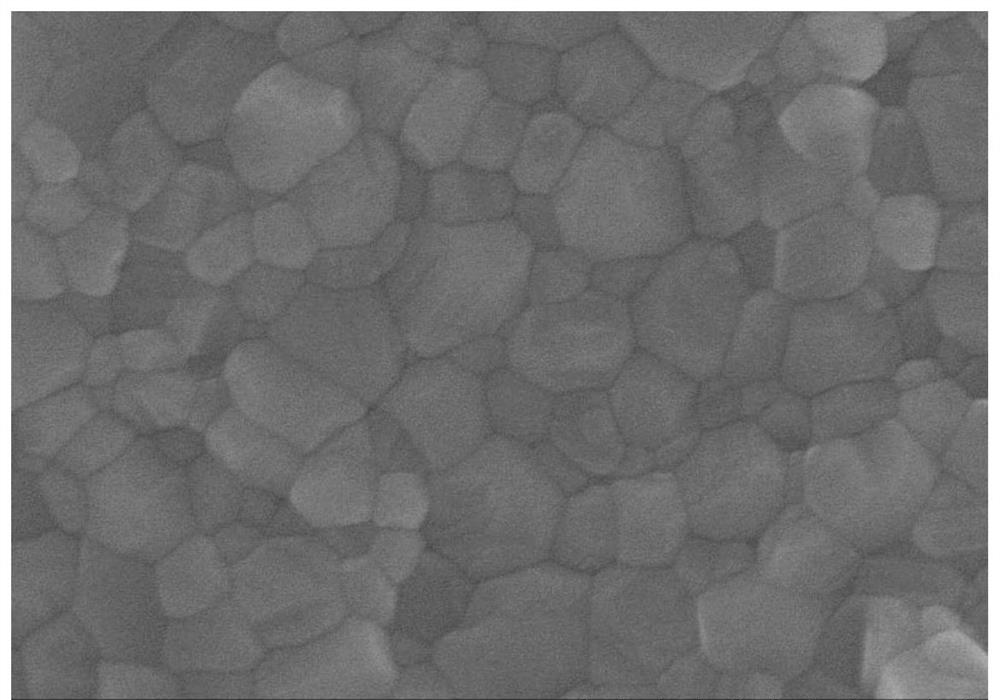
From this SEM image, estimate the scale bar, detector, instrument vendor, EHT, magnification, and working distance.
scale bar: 1000 nm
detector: SE(U)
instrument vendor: Hitachi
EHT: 10 kV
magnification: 50 K X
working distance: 8.7 mm